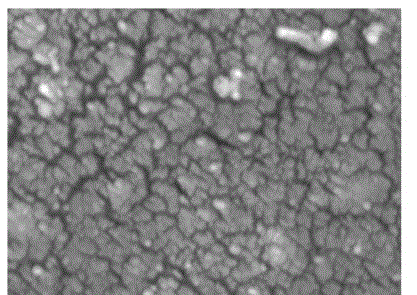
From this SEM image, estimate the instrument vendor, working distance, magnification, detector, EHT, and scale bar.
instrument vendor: JEOL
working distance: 11.6 mm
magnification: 20 K X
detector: LEI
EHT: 15 kV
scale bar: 1000 nm